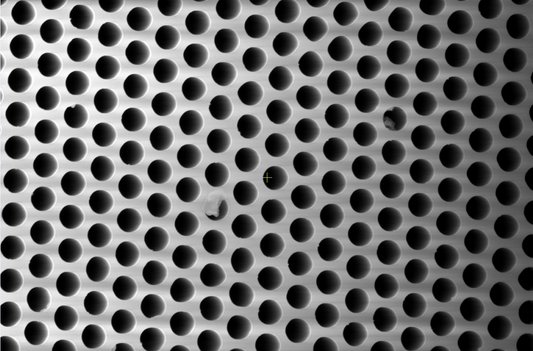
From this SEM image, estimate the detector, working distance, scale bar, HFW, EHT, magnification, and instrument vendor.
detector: ETD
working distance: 20.3 mm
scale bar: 10000 nm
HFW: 21.2 µm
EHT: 20 kV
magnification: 6 K X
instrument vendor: Thermo